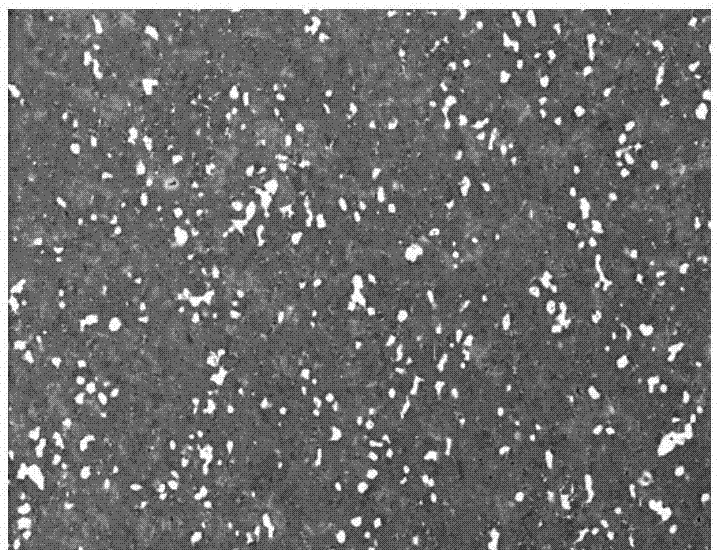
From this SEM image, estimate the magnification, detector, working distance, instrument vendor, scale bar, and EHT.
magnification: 1.1 K X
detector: SE(M)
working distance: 9.2 mm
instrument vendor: Hitachi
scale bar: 50000 nm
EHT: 15 kV